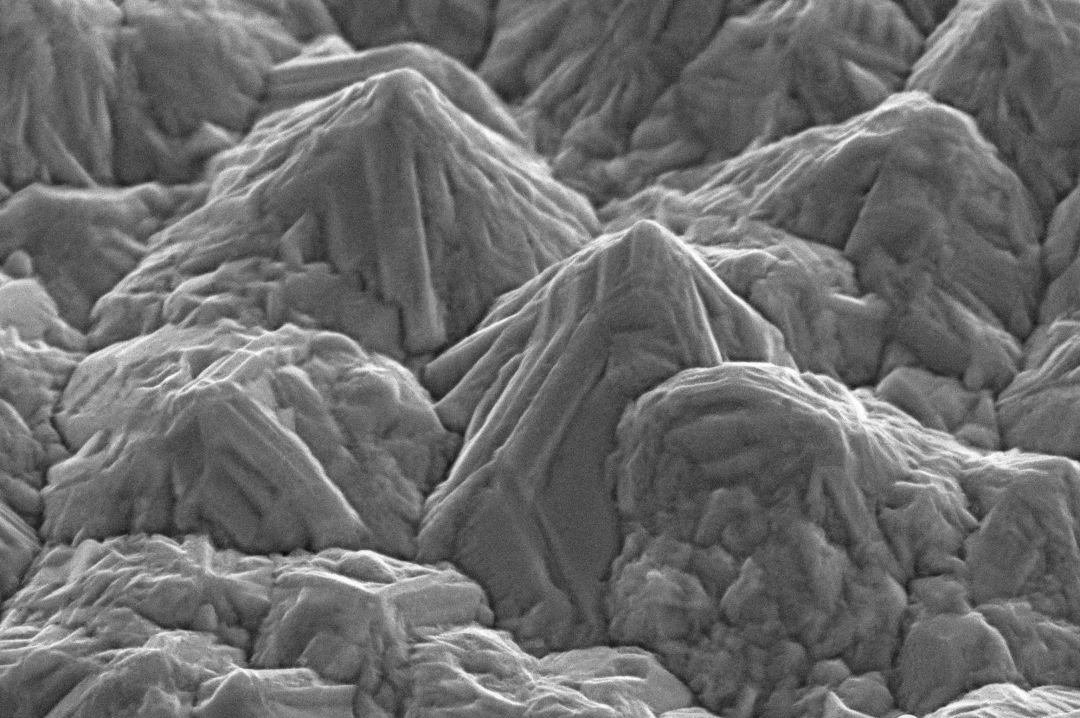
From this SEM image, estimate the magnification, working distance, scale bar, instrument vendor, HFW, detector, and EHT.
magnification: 10 K X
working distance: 3.61 mm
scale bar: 1000 nm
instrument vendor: other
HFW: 12.81 µm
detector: ETD SE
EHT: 10 kV